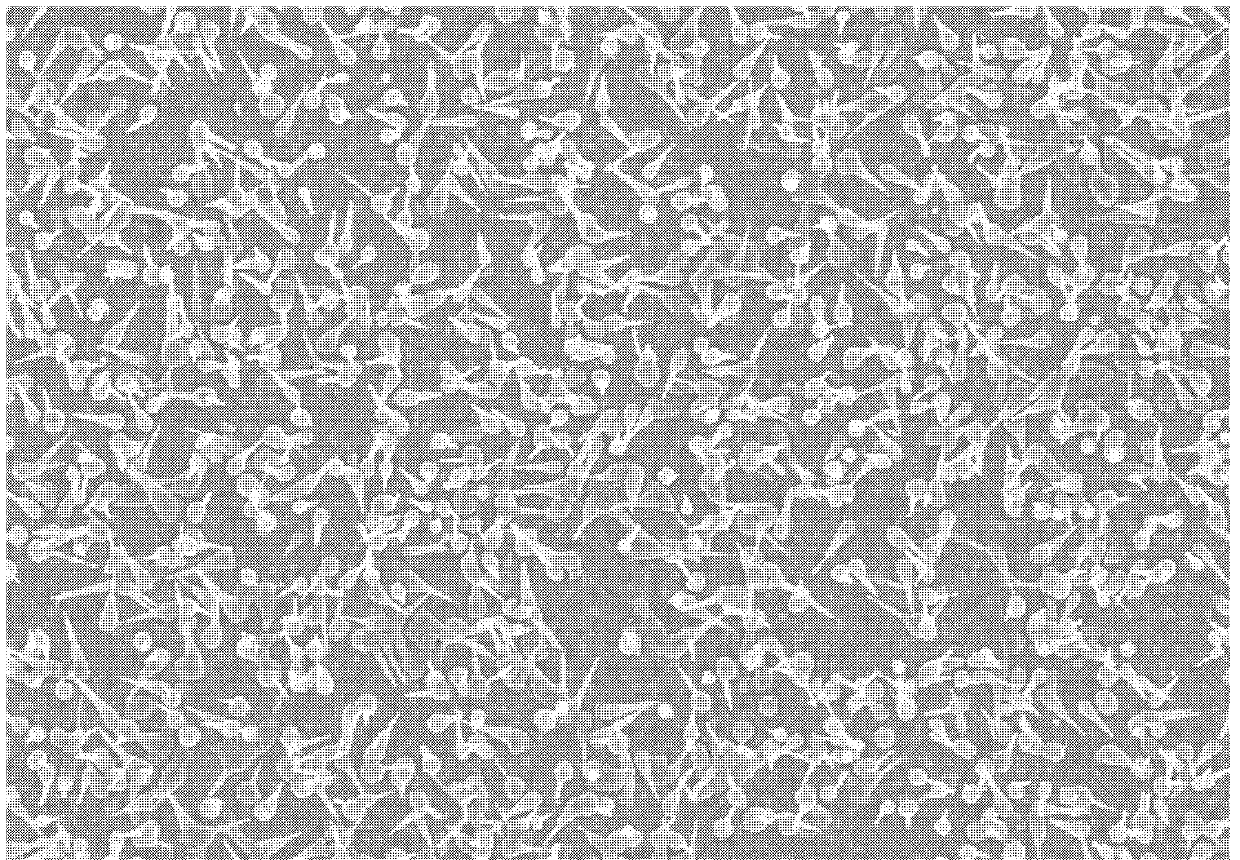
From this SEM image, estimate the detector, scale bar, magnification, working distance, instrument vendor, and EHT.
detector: SE(M)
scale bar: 20000 nm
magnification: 2.5 K X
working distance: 8.4 mm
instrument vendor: Hitachi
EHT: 10 kV